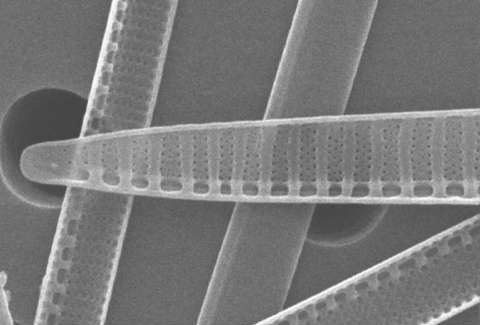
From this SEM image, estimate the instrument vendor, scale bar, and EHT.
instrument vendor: JEOL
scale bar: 1000 nm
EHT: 25 kV